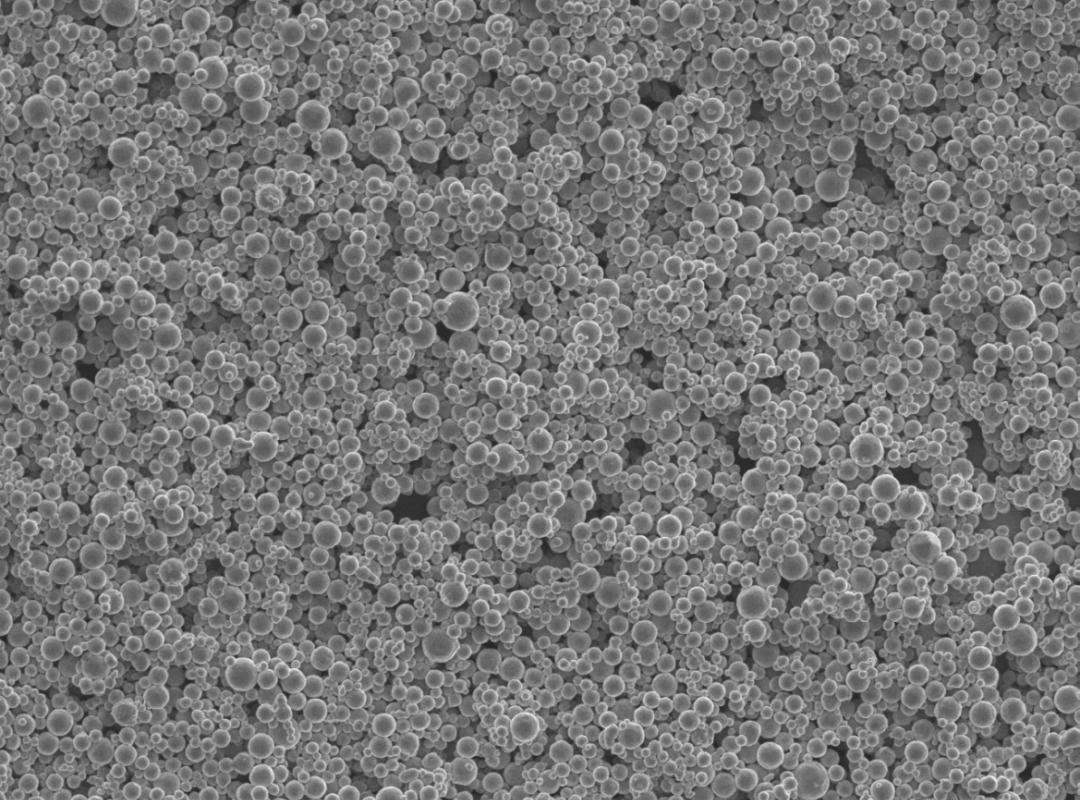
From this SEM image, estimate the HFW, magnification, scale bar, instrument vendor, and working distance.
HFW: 4.23 µm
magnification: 30 K X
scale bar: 200 nm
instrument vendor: Phenom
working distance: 2.08 mm